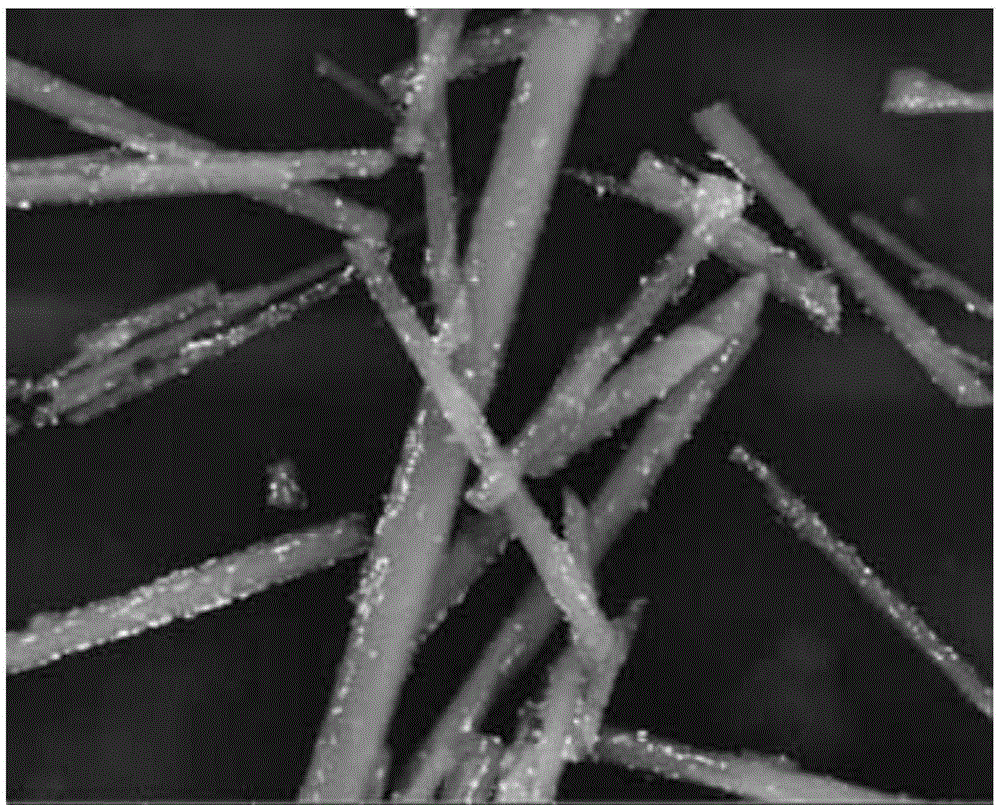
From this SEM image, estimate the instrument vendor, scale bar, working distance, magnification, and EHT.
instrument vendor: FEI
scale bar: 10000 nm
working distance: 11.2 mm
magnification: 10 K X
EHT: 20 kV